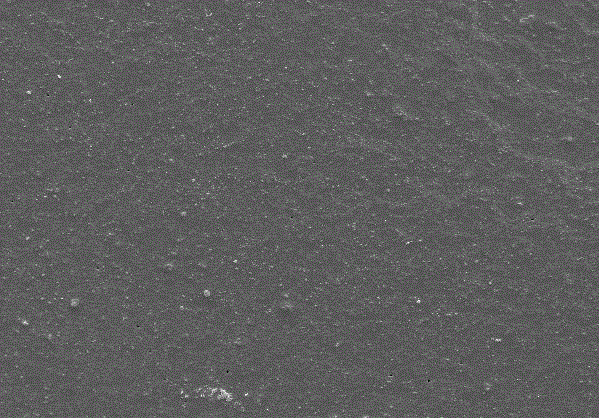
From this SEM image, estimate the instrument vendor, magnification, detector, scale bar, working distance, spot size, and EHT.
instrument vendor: FEI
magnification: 0.5 K X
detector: SE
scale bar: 50000 nm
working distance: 5.1 mm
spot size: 3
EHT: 20 kV